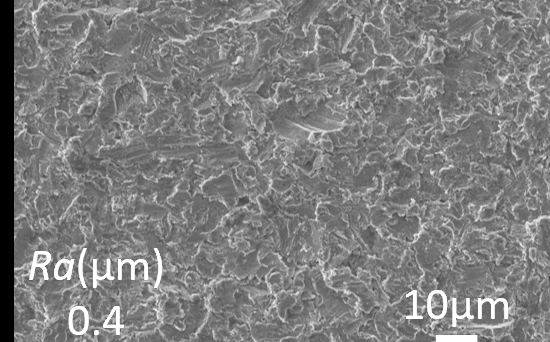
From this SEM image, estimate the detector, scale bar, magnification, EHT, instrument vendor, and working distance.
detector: SED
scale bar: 10000 nm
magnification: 1 K X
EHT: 10 kV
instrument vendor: JEOL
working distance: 11.6 mm